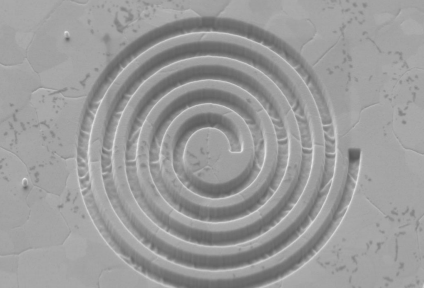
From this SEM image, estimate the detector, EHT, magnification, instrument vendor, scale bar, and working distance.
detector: SE2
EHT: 5 kV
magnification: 12.93 K X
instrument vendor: Zeiss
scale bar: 300 nm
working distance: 5.1 mm